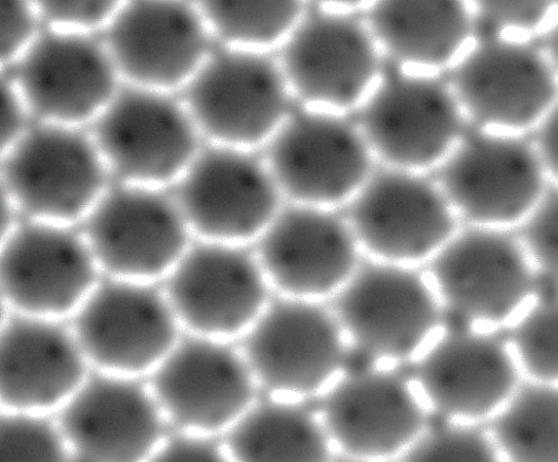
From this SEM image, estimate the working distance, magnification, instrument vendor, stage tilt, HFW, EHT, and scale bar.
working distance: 10.8 mm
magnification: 150 K X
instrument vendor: FEI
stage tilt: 0°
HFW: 1.8 µm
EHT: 25 kV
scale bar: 500 nm